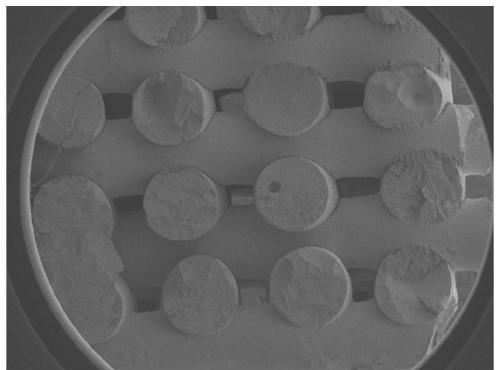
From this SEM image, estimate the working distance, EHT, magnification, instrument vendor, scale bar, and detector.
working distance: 23 mm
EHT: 5 kV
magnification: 0.025 K X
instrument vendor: JEOL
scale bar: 1000000 nm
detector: SEI